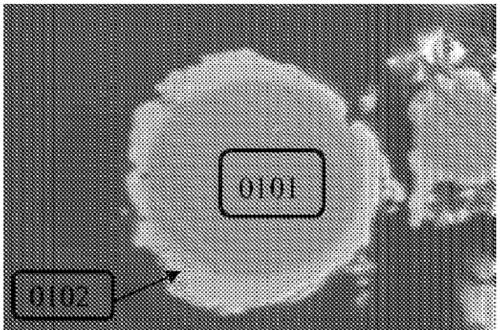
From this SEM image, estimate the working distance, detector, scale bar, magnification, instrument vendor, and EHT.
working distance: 13.8 mm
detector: SE(M)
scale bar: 20000 nm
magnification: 2 K X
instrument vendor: Hitachi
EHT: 15 kV